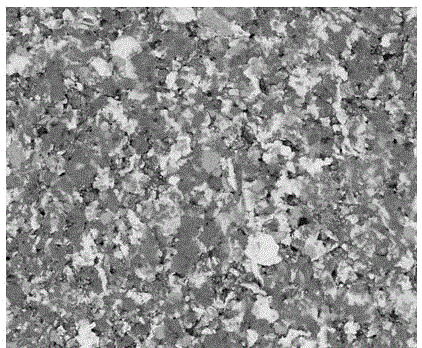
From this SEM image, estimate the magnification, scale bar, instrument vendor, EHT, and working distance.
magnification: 0.5 K X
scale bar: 300000 nm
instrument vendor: FEI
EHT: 15 kV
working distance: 10 mm